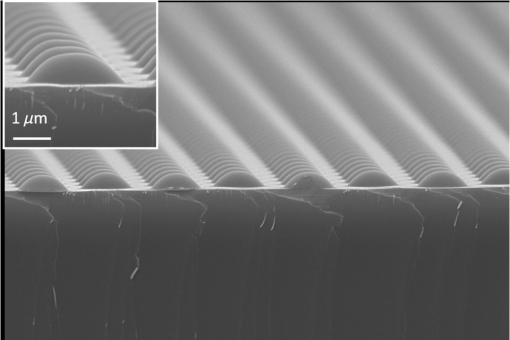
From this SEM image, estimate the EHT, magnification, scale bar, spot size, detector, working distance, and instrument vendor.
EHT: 20 kV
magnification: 5 K X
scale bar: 5000 nm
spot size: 40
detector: SEI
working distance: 11 mm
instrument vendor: JEOL